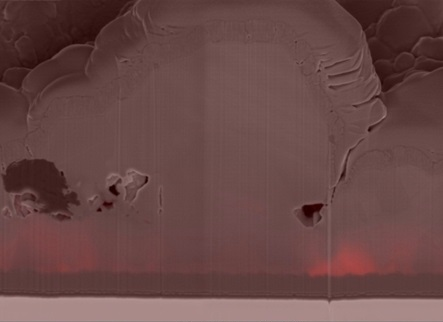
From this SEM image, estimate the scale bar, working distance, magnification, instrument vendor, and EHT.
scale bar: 2000 nm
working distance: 5.1 mm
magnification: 30 K X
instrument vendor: Zeiss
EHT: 3 kV